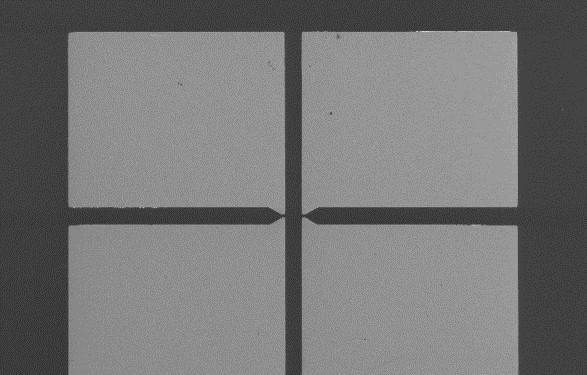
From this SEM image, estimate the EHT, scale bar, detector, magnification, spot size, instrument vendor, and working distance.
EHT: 5 kV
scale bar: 500000 nm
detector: SE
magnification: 0.069 K X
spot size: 3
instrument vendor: FEI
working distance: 6.9 mm